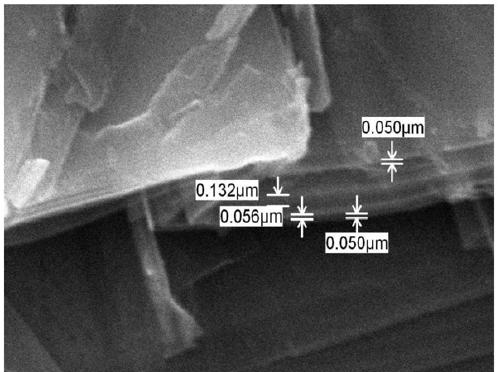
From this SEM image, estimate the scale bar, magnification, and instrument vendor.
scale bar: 1000 nm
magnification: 20 K X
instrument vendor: JEOL